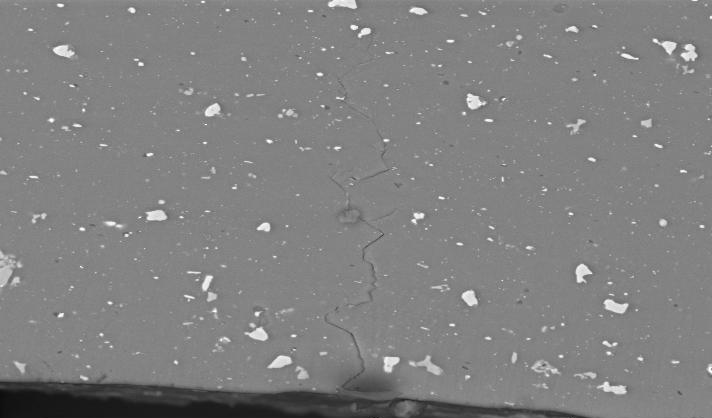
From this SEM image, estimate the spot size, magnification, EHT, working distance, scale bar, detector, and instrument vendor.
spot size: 5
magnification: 0.5 K X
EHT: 20 kV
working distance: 10.7 mm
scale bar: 50000 nm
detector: BSE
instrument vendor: FEI